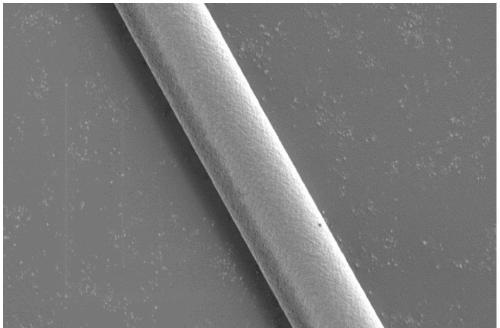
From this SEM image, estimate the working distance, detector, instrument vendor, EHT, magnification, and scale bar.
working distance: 4.7 mm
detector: InLens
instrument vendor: Zeiss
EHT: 1 kV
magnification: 3 K X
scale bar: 2000 nm